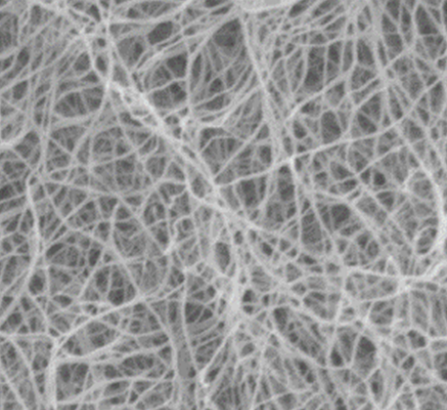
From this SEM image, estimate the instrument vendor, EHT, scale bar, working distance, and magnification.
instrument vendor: JEOL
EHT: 1.5 kV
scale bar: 100 nm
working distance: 4.1 mm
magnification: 50 K X